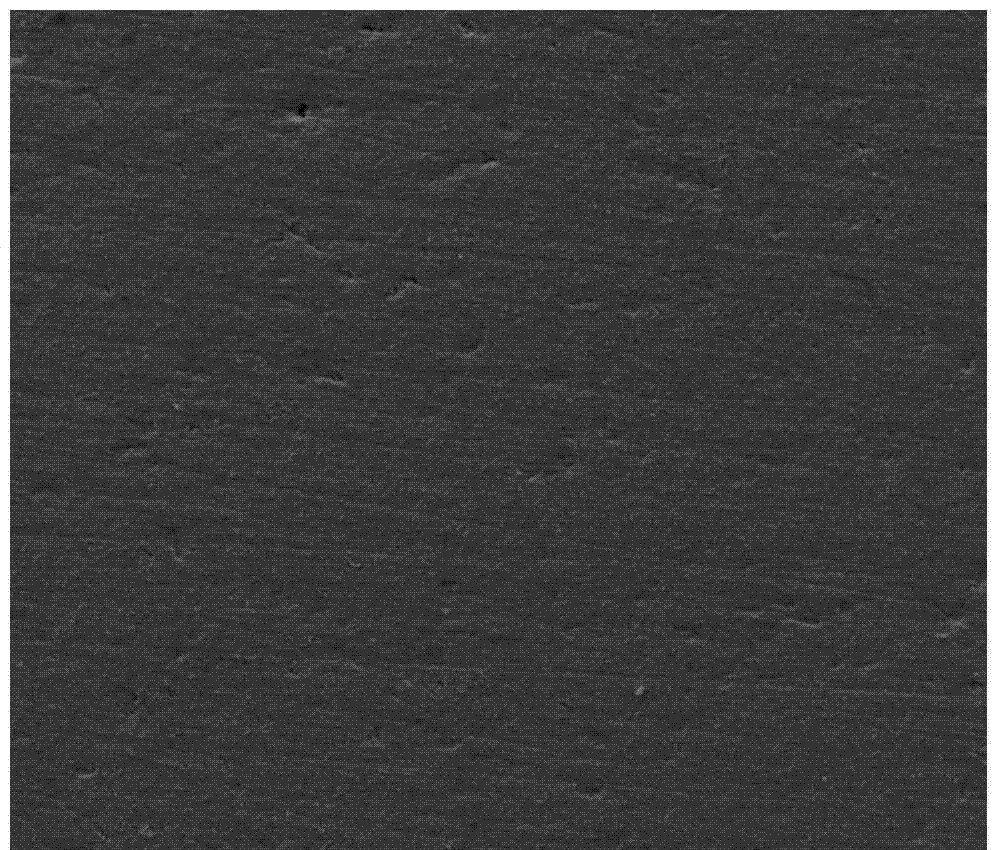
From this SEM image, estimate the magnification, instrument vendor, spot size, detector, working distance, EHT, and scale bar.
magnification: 0.5 K X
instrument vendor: FEI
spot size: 4.5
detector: ETD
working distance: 12.4 mm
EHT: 15 kV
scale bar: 200000 nm